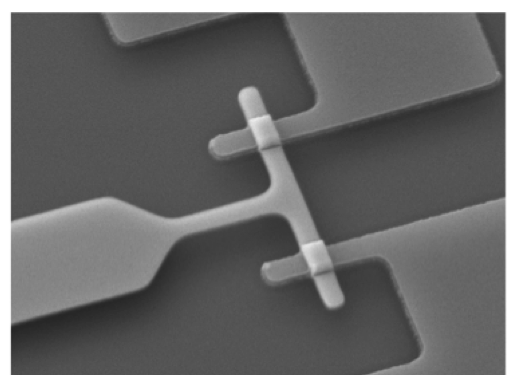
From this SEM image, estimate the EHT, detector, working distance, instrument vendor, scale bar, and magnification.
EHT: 15 kV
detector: LEI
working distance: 20.5 mm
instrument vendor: JEOL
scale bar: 1000 nm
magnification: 8 K X